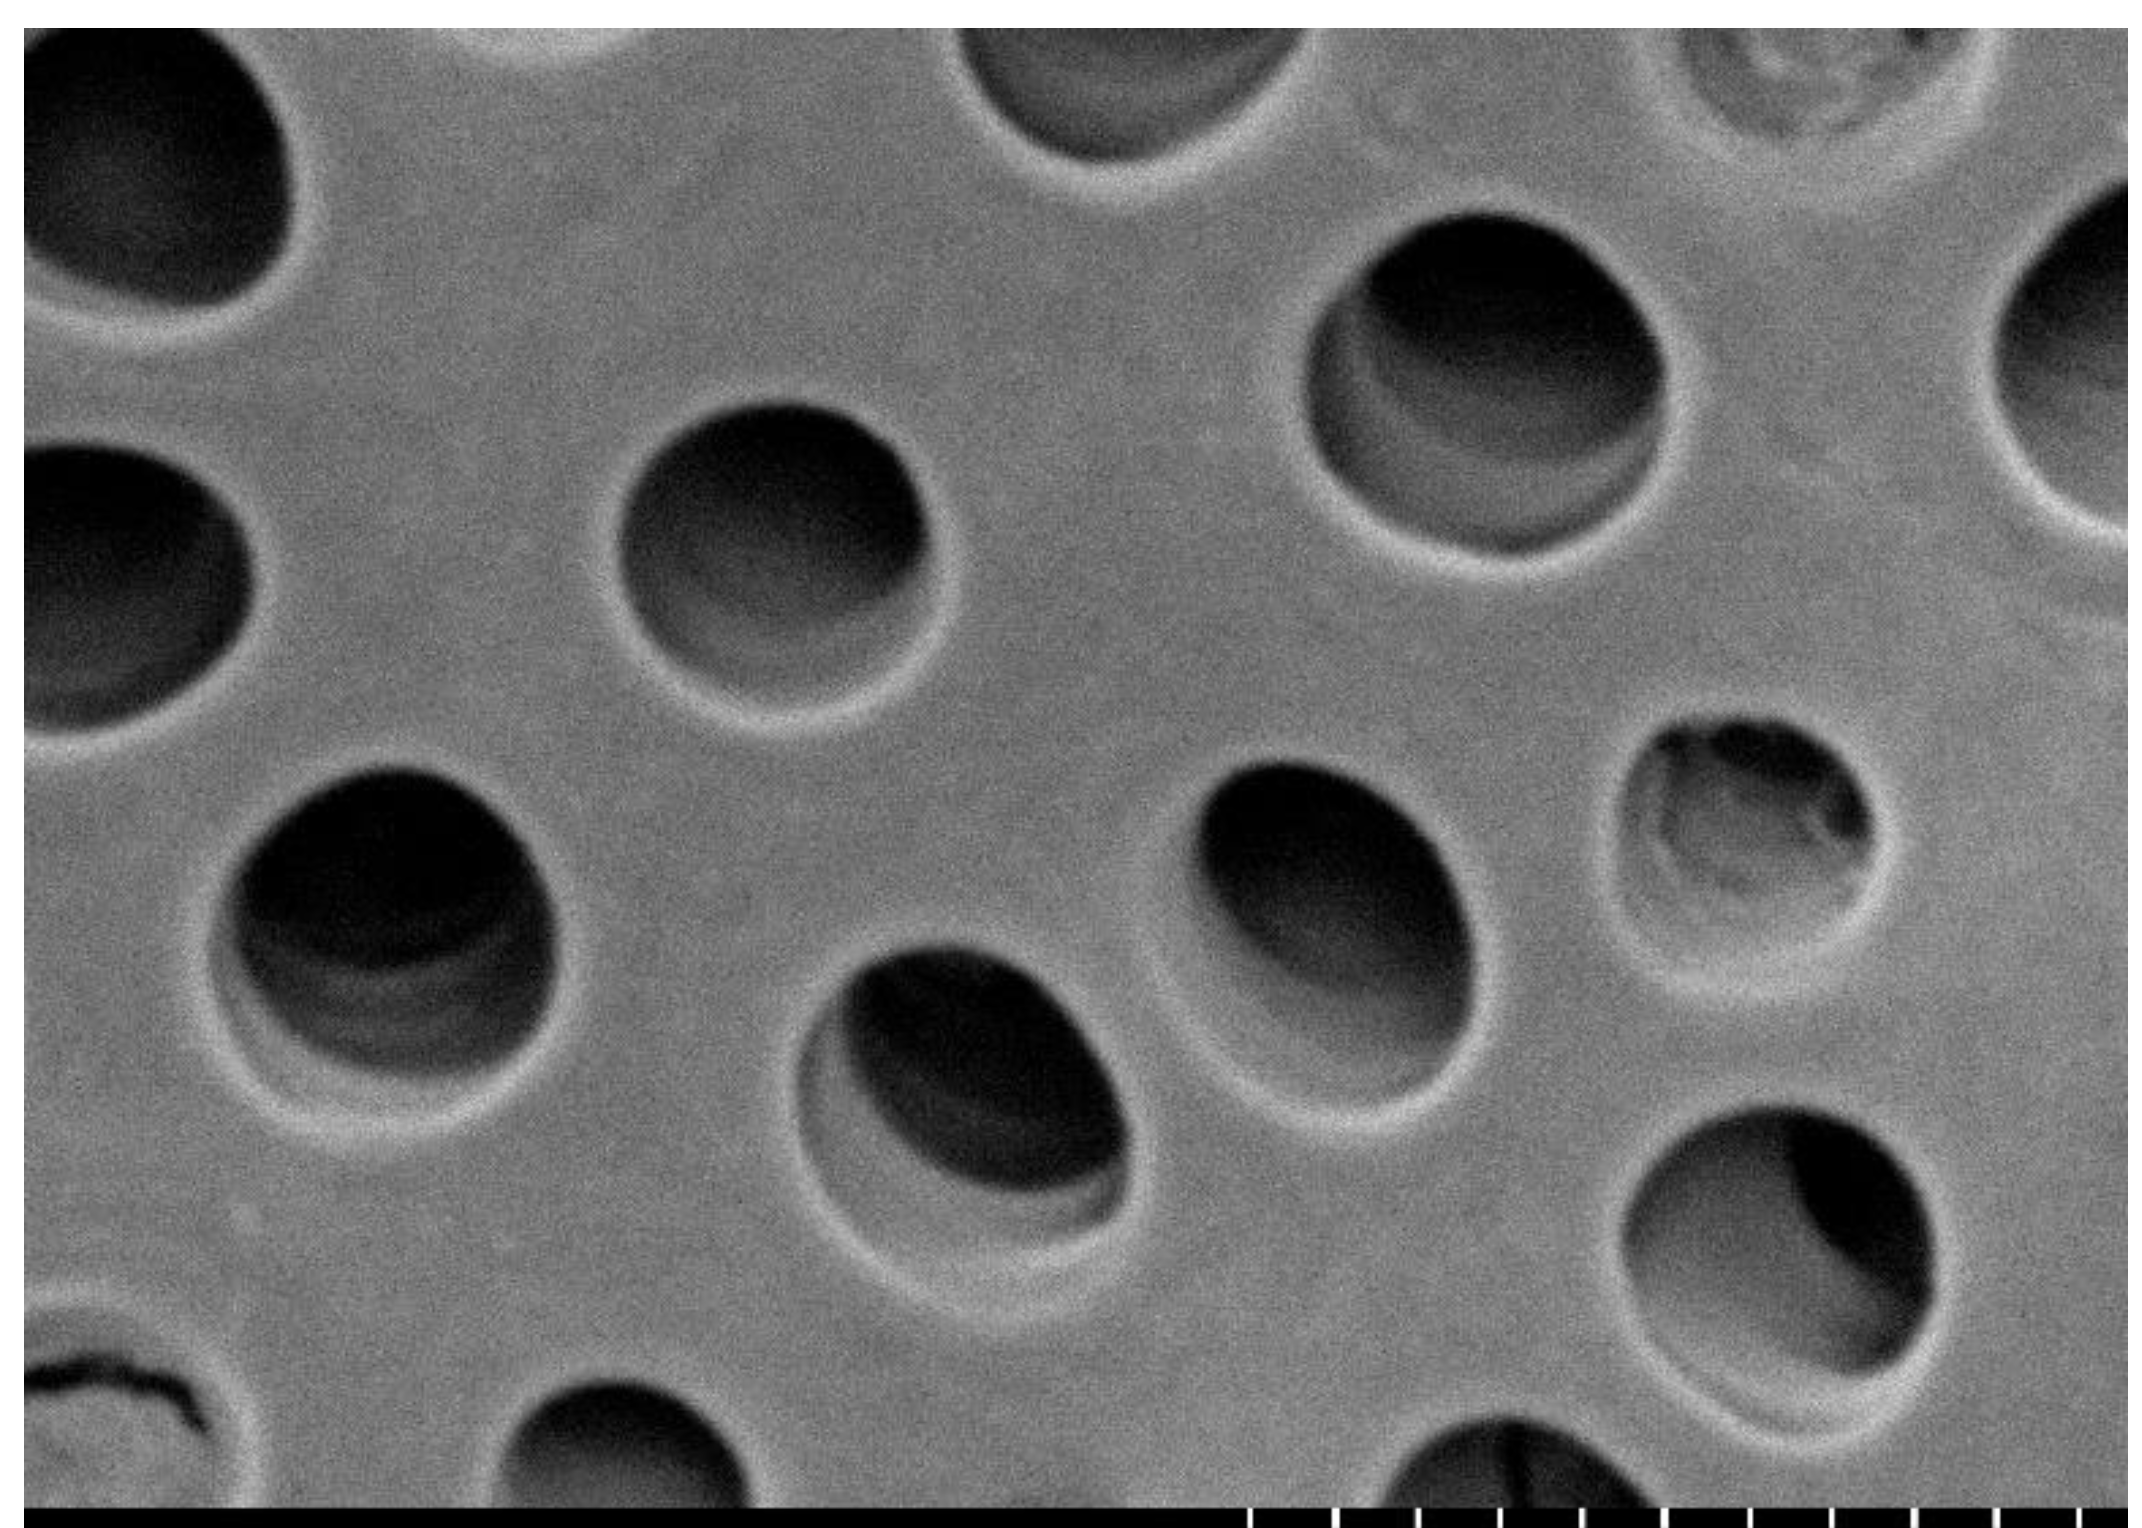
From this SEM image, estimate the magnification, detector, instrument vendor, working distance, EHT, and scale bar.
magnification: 5 K X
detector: Mix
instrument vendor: Hitachi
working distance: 9.7 mm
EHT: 5 kV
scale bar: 10000 nm